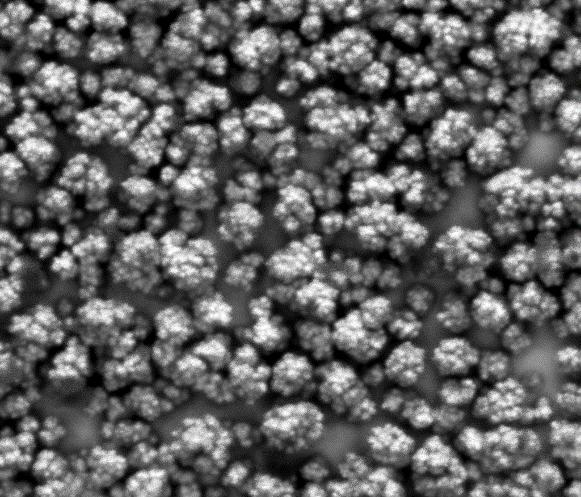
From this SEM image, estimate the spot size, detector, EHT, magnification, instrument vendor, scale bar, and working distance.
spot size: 5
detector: Mix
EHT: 20 kV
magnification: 1 K X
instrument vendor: FEI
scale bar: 50000 nm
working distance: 10.9 mm